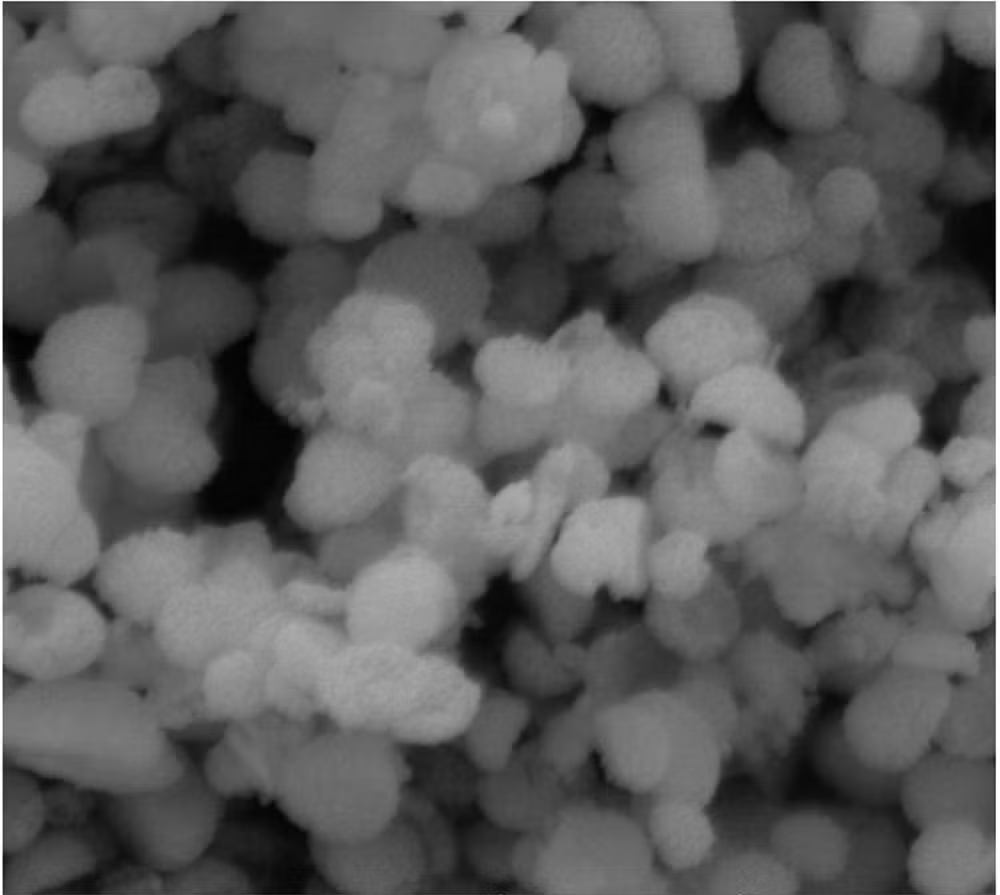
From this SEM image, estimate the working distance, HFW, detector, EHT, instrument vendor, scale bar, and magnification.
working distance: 11.72 mm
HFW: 4.15 µm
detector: SE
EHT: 10 kV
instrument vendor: Tescan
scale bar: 1000 nm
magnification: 50 K X